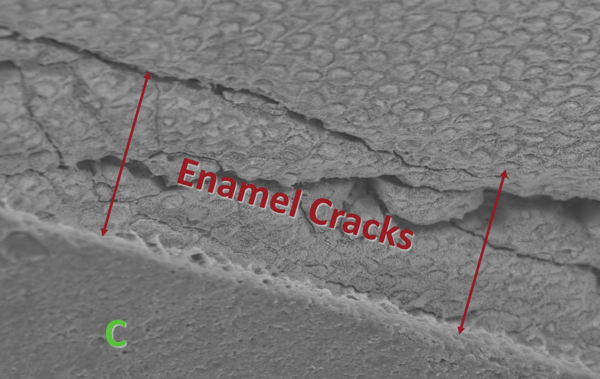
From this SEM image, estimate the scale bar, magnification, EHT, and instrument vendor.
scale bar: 10000 nm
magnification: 1 K X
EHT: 10 kV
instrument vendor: JEOL